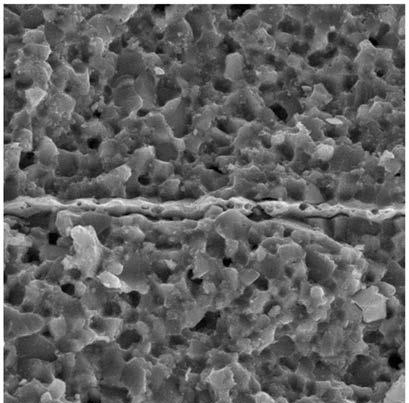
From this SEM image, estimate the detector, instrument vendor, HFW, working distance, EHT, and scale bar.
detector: SE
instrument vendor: Tescan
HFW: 56.9 µm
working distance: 11.07 mm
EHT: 30 kV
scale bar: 10000 nm